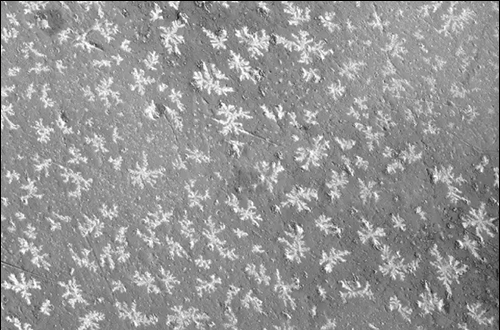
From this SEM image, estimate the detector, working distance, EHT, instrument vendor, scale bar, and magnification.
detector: SE2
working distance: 19.6 mm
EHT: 10 kV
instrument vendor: Zeiss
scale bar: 2000 nm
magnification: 2 K X